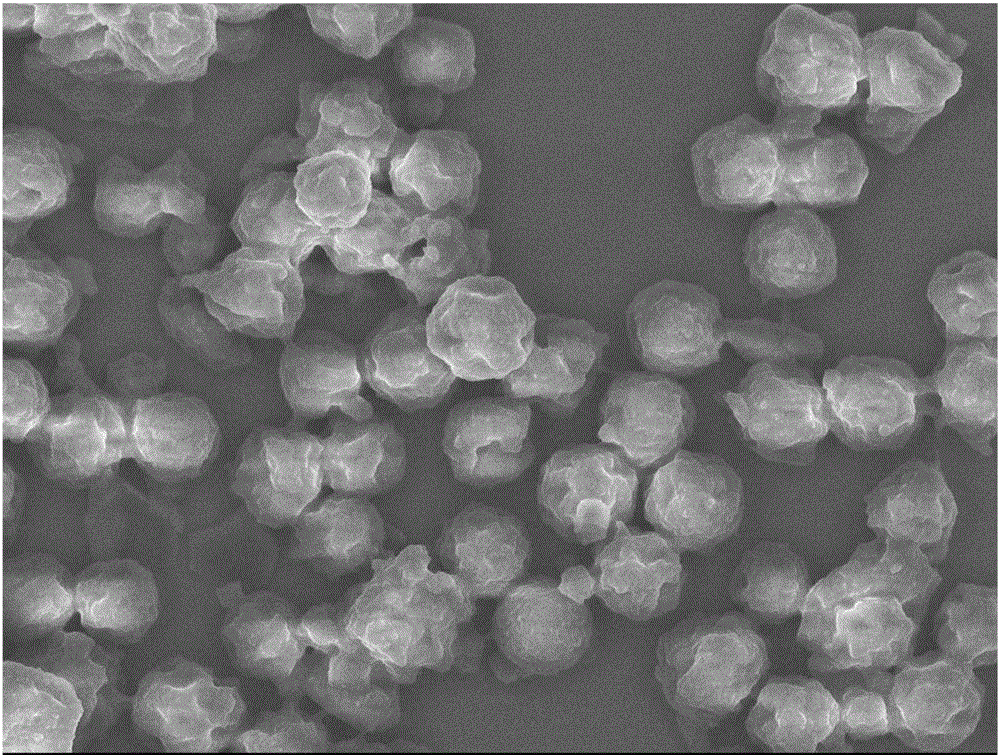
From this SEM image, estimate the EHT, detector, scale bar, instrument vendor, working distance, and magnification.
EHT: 5 kV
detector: SEI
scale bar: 1000 nm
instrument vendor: JEOL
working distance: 6 mm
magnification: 6 K X